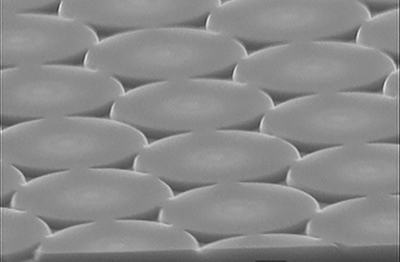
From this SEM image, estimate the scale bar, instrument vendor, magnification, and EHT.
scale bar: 1000 nm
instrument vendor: JEOL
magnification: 10 K X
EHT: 15 kV